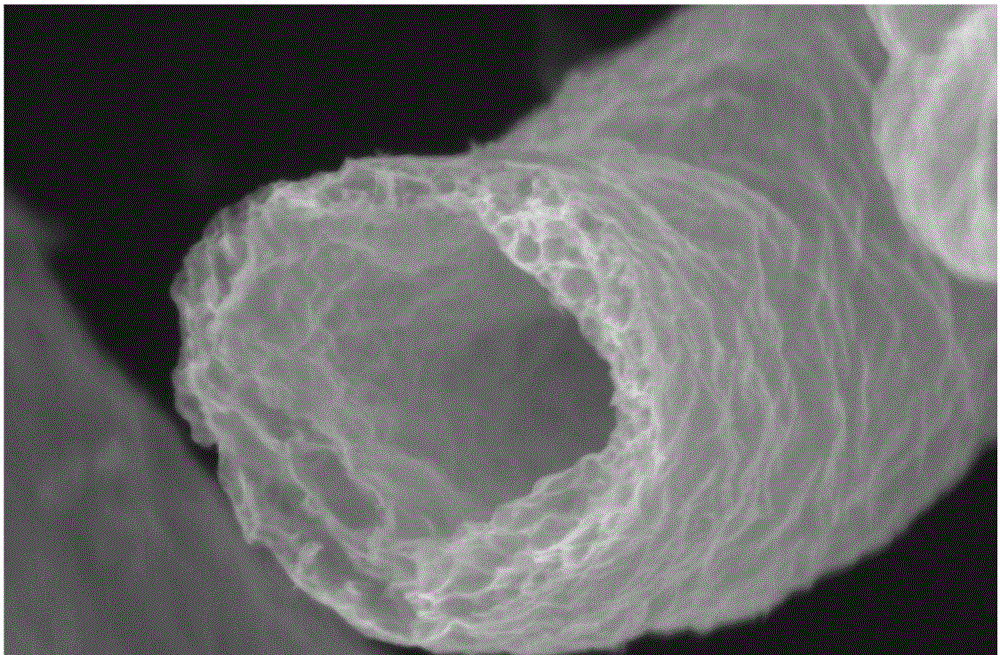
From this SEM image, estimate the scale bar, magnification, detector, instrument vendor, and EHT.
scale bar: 5000 nm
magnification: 5 K X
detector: SEI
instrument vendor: JEOL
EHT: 20 kV